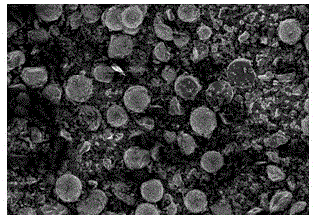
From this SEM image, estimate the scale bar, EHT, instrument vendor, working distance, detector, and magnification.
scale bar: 100000 nm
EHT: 3 kV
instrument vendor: Hitachi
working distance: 8.3 mm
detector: SE(U)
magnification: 0.5 K X